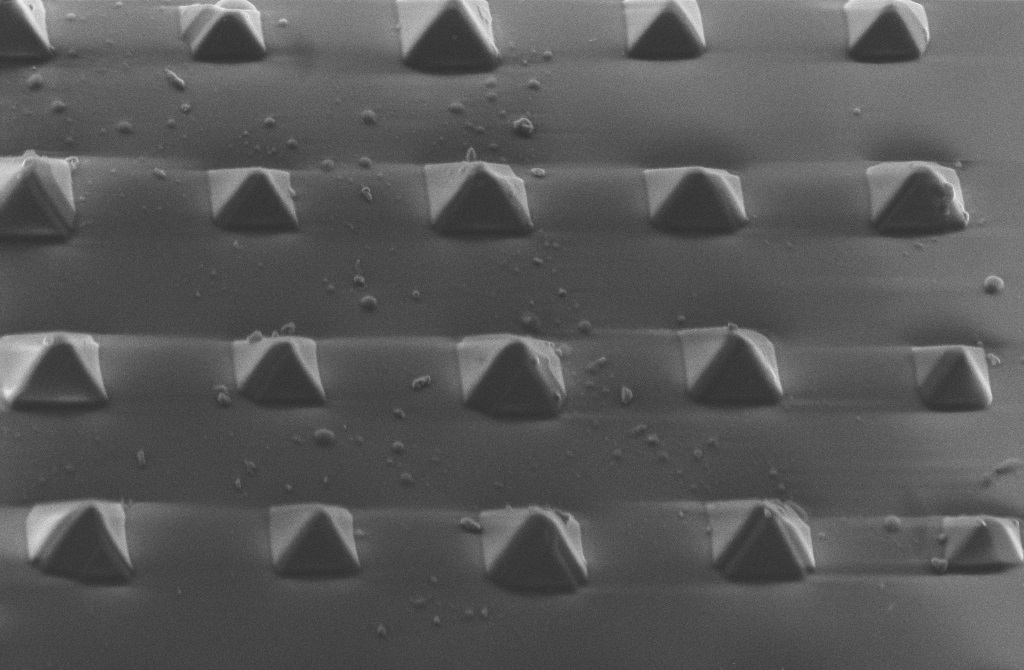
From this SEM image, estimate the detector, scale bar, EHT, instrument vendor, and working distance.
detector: SE2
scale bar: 2000 nm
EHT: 5 kV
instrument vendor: Zeiss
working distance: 5.3 mm